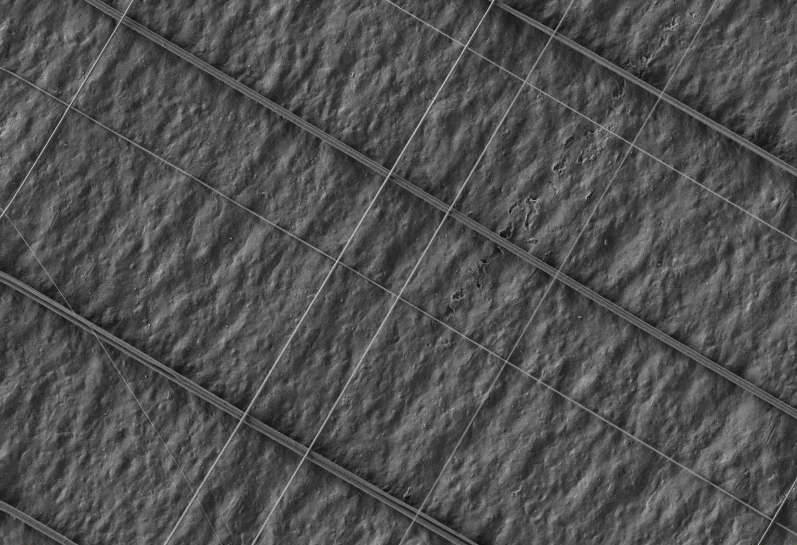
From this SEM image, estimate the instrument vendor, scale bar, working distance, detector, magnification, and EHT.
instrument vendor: Zeiss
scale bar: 20000 nm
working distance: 3.7 mm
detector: SE2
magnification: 0.5 K X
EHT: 5 kV